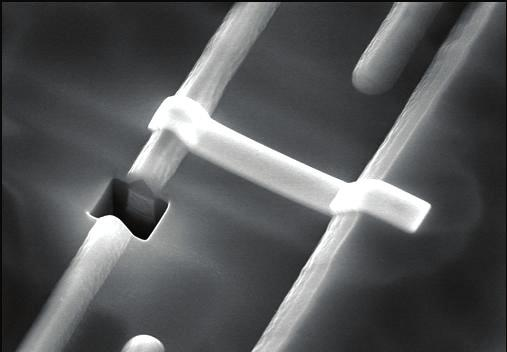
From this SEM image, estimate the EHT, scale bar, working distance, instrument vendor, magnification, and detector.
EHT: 15 kV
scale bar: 5000 nm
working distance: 8.1 mm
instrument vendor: Hitachi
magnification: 11 K X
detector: SE(M)